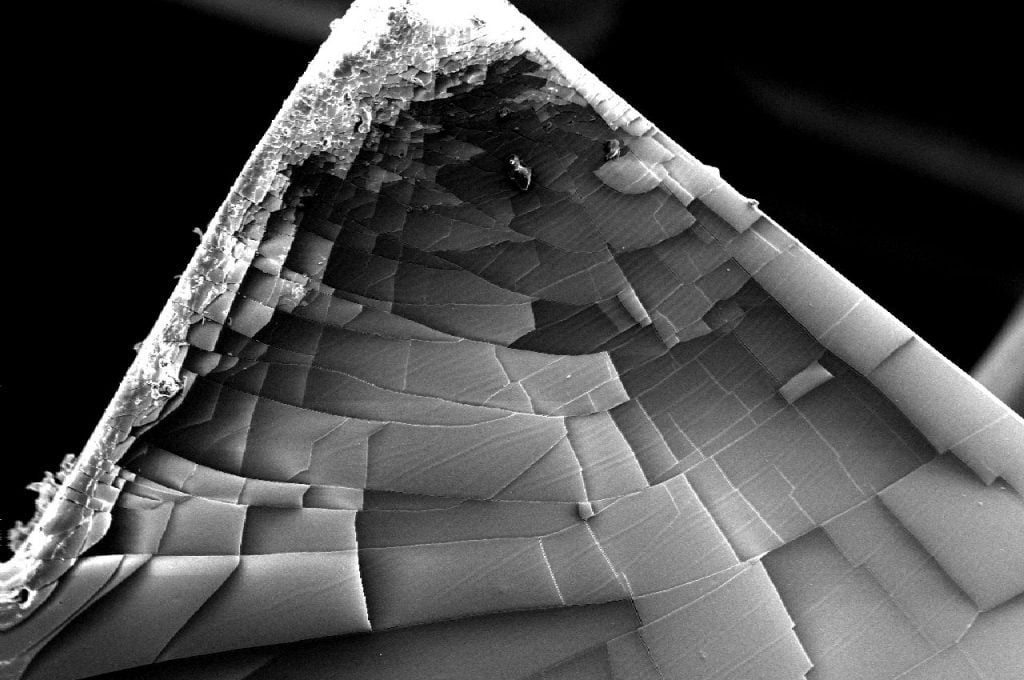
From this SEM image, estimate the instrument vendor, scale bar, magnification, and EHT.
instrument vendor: JEOL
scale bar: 100000 nm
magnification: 0.1 K X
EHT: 10 kV